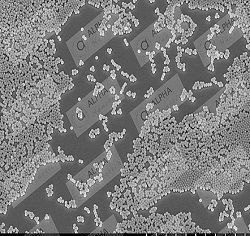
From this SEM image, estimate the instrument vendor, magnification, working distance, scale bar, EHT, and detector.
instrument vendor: Hitachi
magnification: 10 K X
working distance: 12.2 mm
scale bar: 5000 nm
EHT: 5 kV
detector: SE(U)